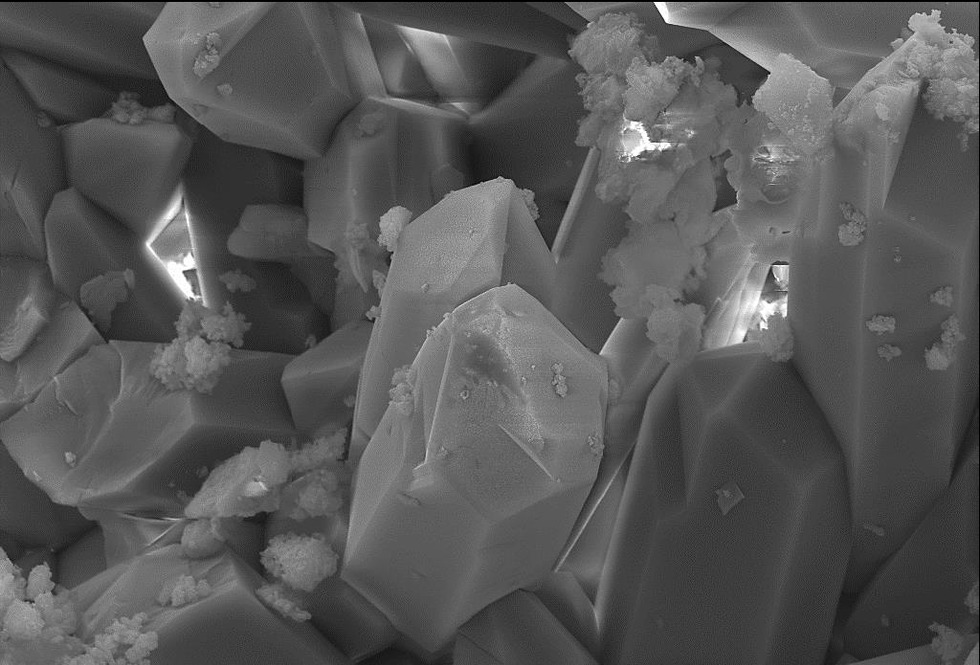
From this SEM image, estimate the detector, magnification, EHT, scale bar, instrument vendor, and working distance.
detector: SE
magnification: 2.5 K X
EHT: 20 kV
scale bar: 10000 nm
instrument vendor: other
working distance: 10.83 mm